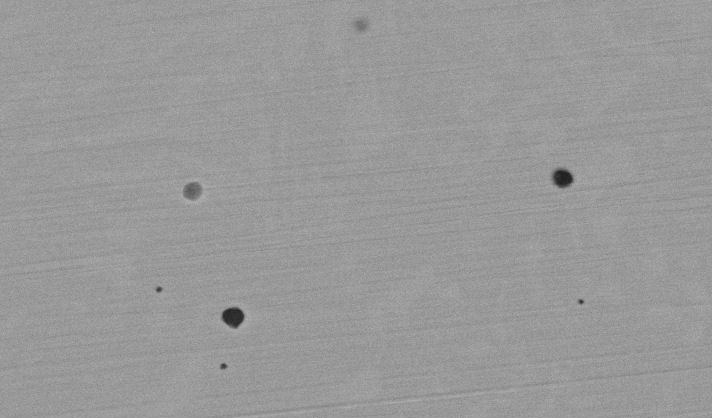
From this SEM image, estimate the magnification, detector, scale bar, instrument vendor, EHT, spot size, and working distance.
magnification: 5 K X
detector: BSE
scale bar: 10000 nm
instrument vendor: FEI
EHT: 20 kV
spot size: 4.5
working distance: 10.4 mm